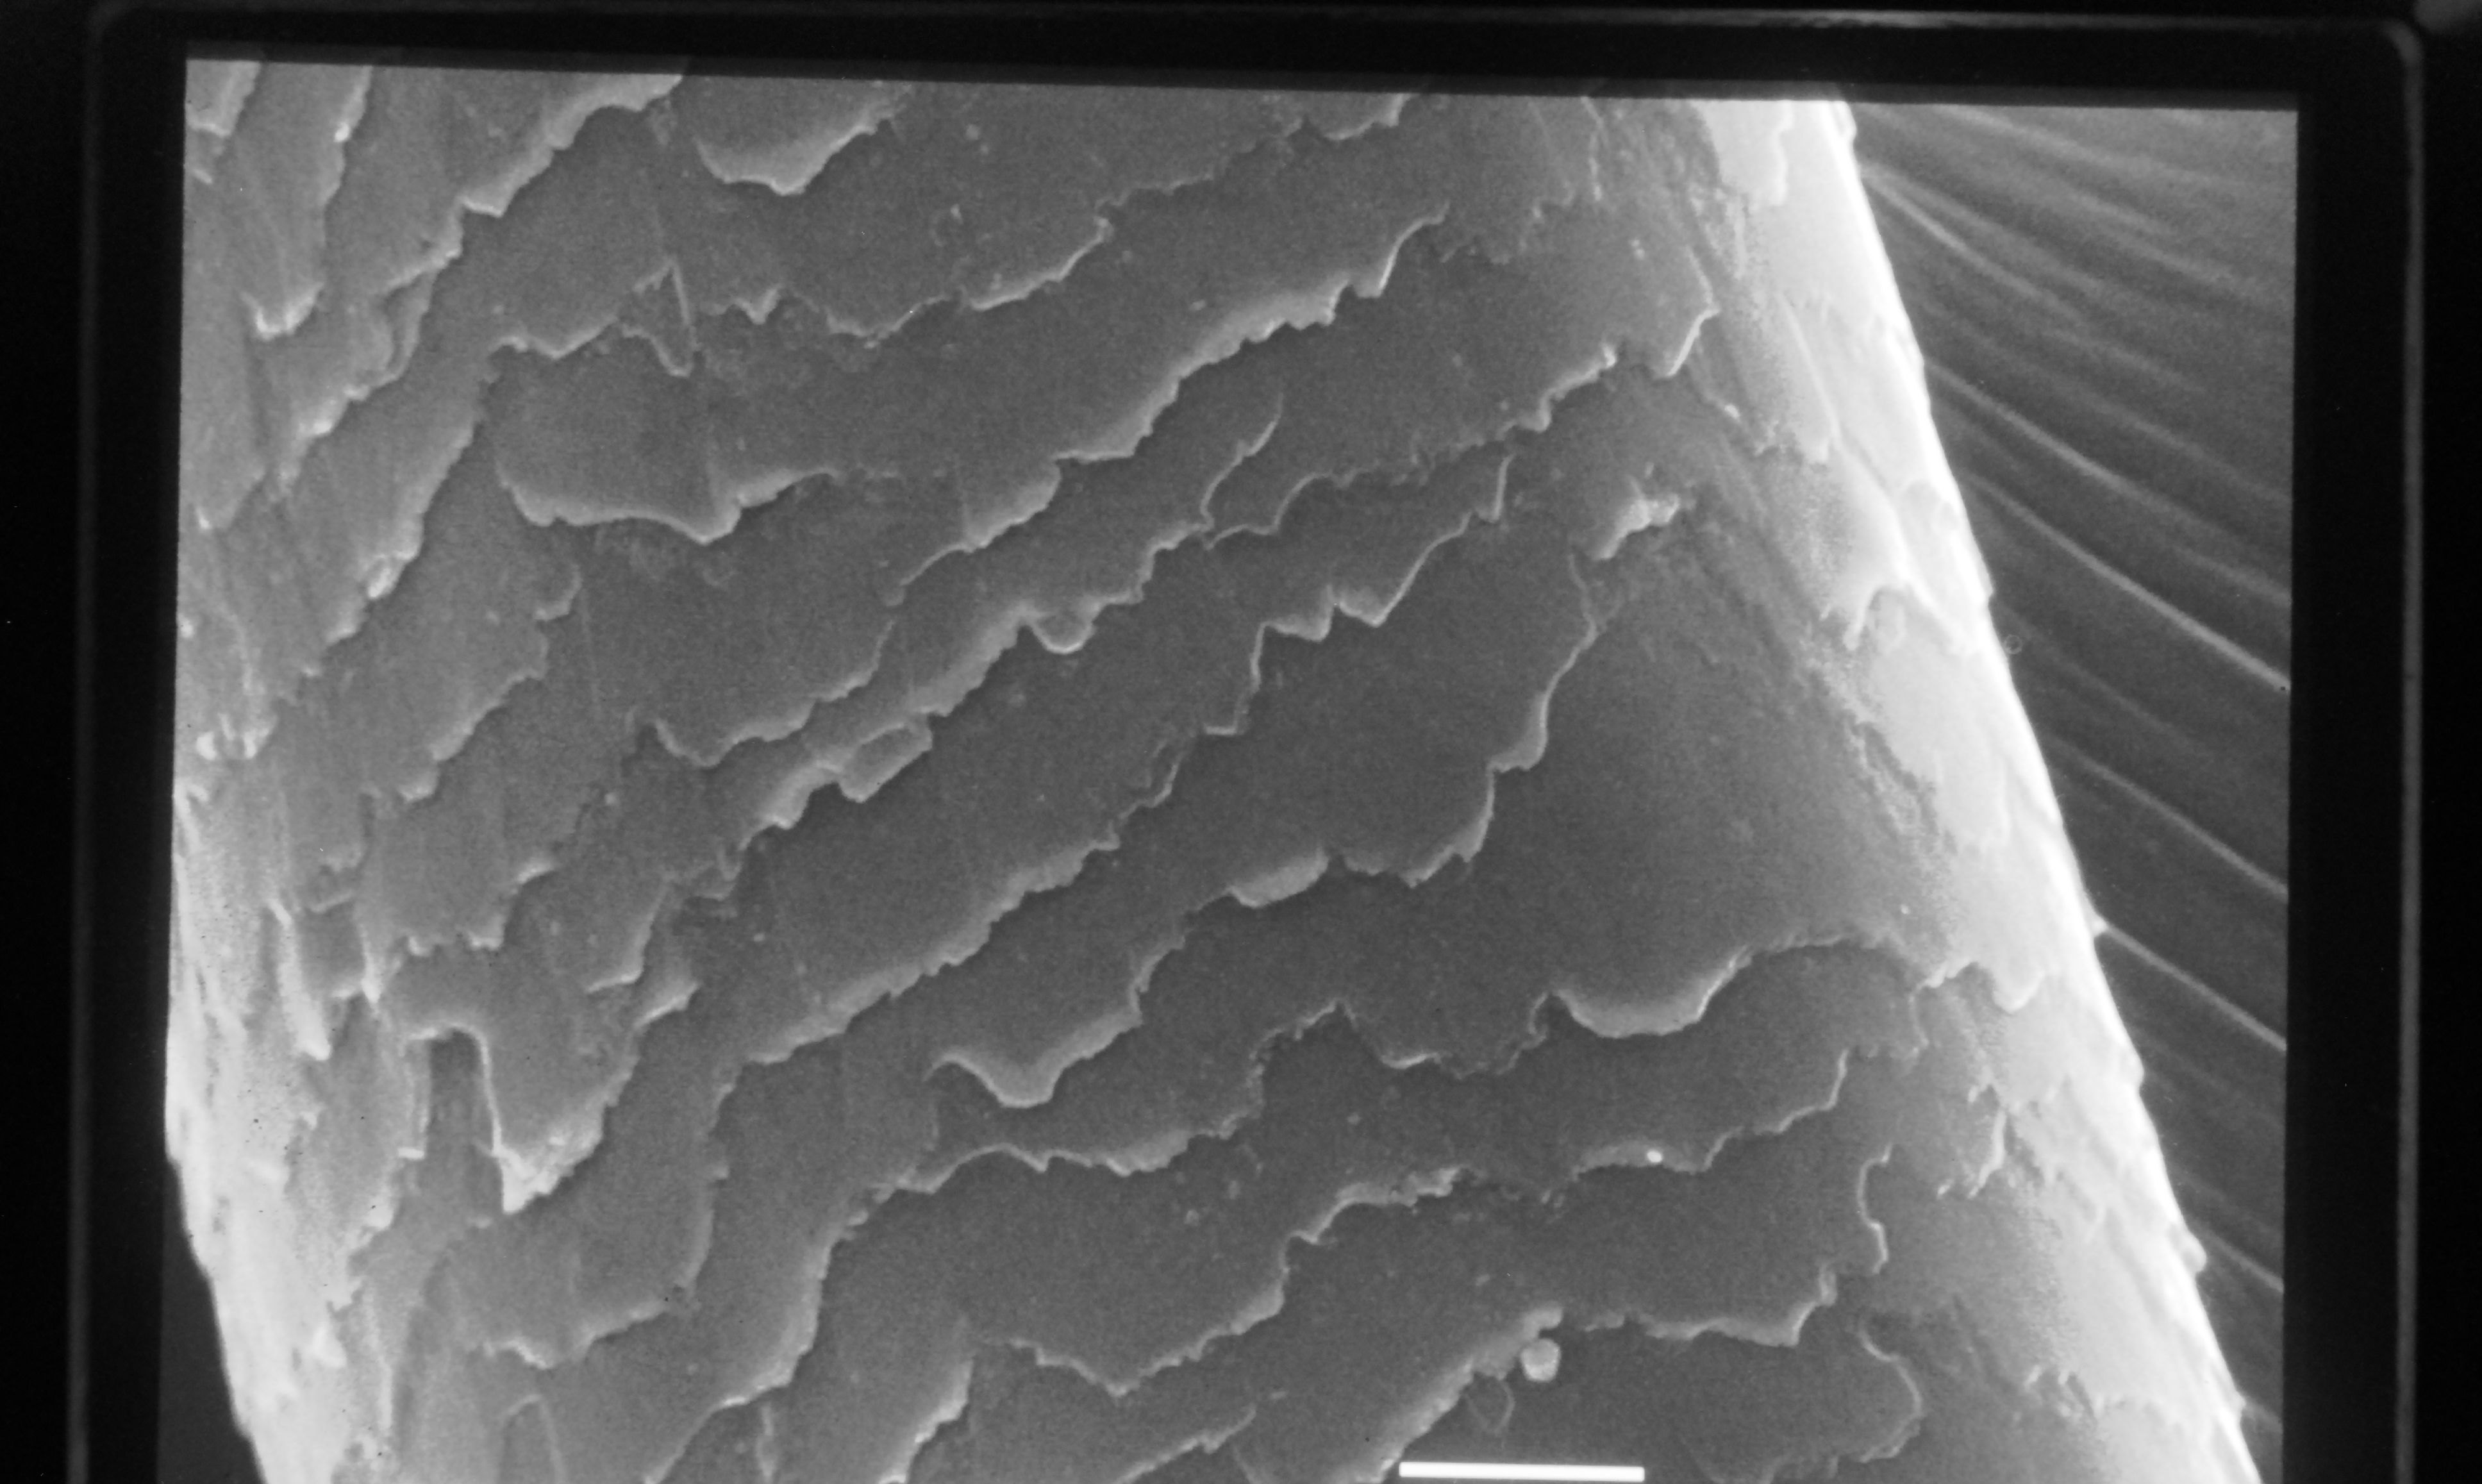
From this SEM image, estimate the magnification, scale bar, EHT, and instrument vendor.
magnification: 1.5 K X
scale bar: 10000 nm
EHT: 25 kV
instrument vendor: JEOL